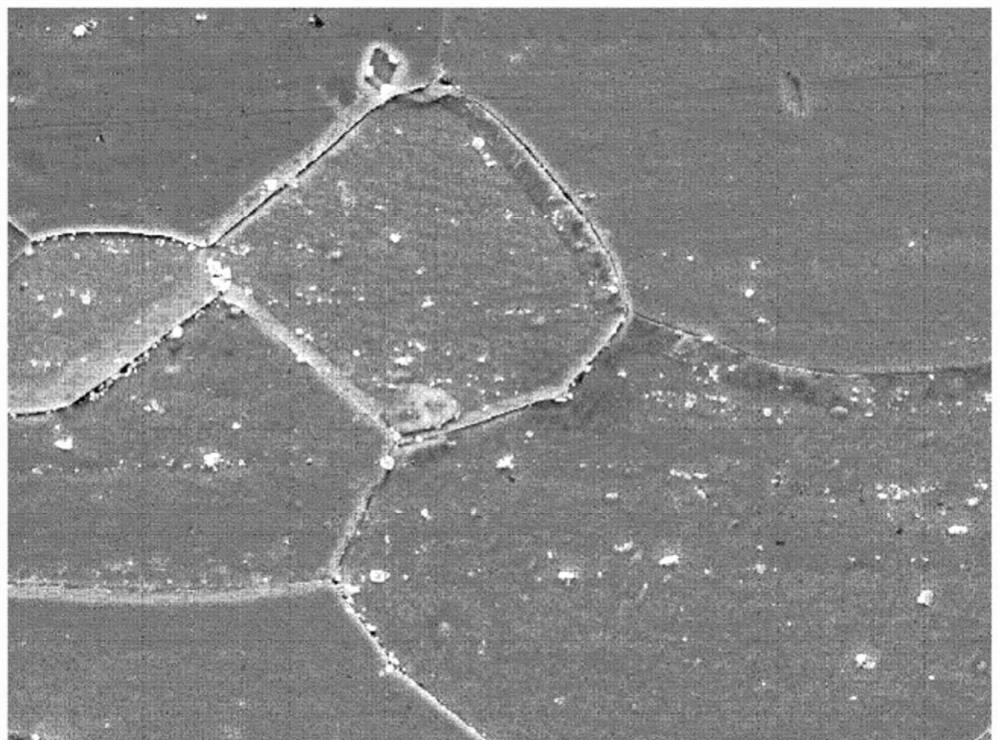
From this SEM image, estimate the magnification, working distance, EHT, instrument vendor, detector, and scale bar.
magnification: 2 K X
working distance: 10 mm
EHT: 15 kV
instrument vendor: JEOL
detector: SEI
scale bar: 10000 nm